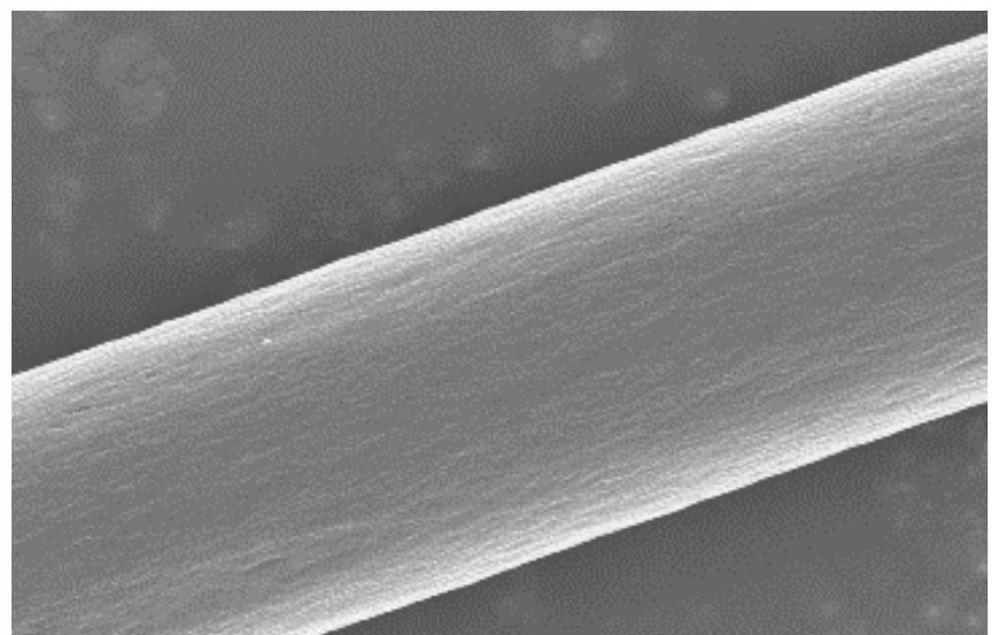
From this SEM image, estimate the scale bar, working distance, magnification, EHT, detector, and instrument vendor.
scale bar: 3000 nm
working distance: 7.7 mm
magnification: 15 K X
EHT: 5 kV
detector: SE(M)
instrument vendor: Hitachi